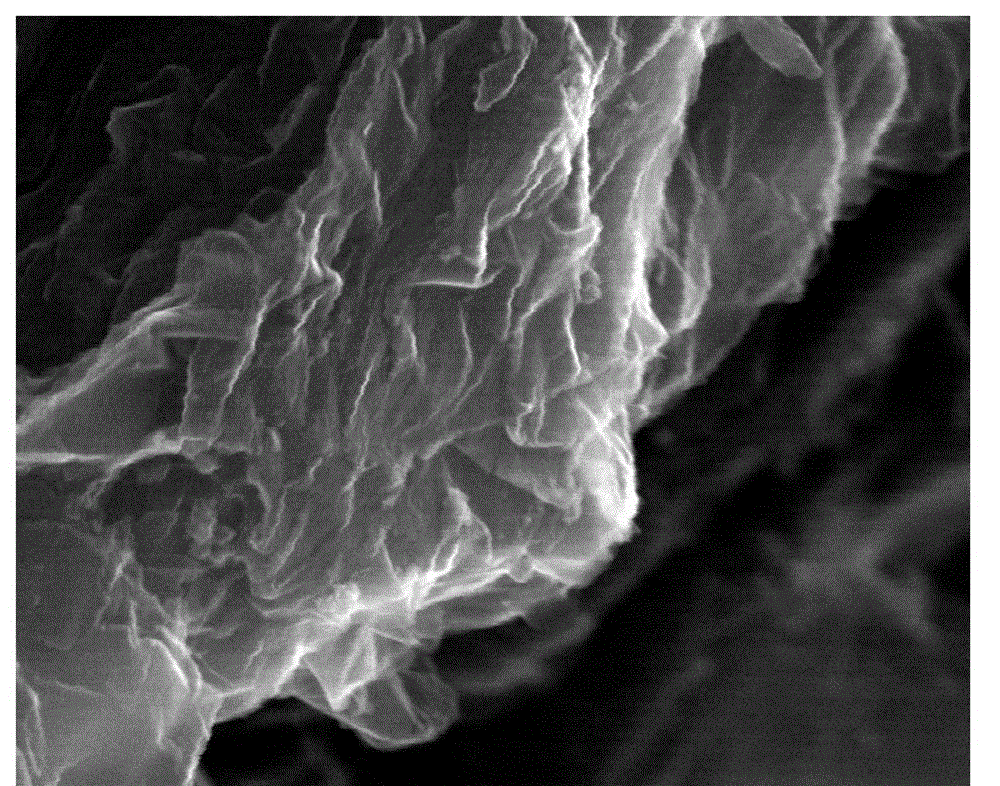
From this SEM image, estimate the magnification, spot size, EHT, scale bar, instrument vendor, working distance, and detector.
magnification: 100 K X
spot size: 3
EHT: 20 kV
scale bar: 500 nm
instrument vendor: FEI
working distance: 10.2 mm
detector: ETD